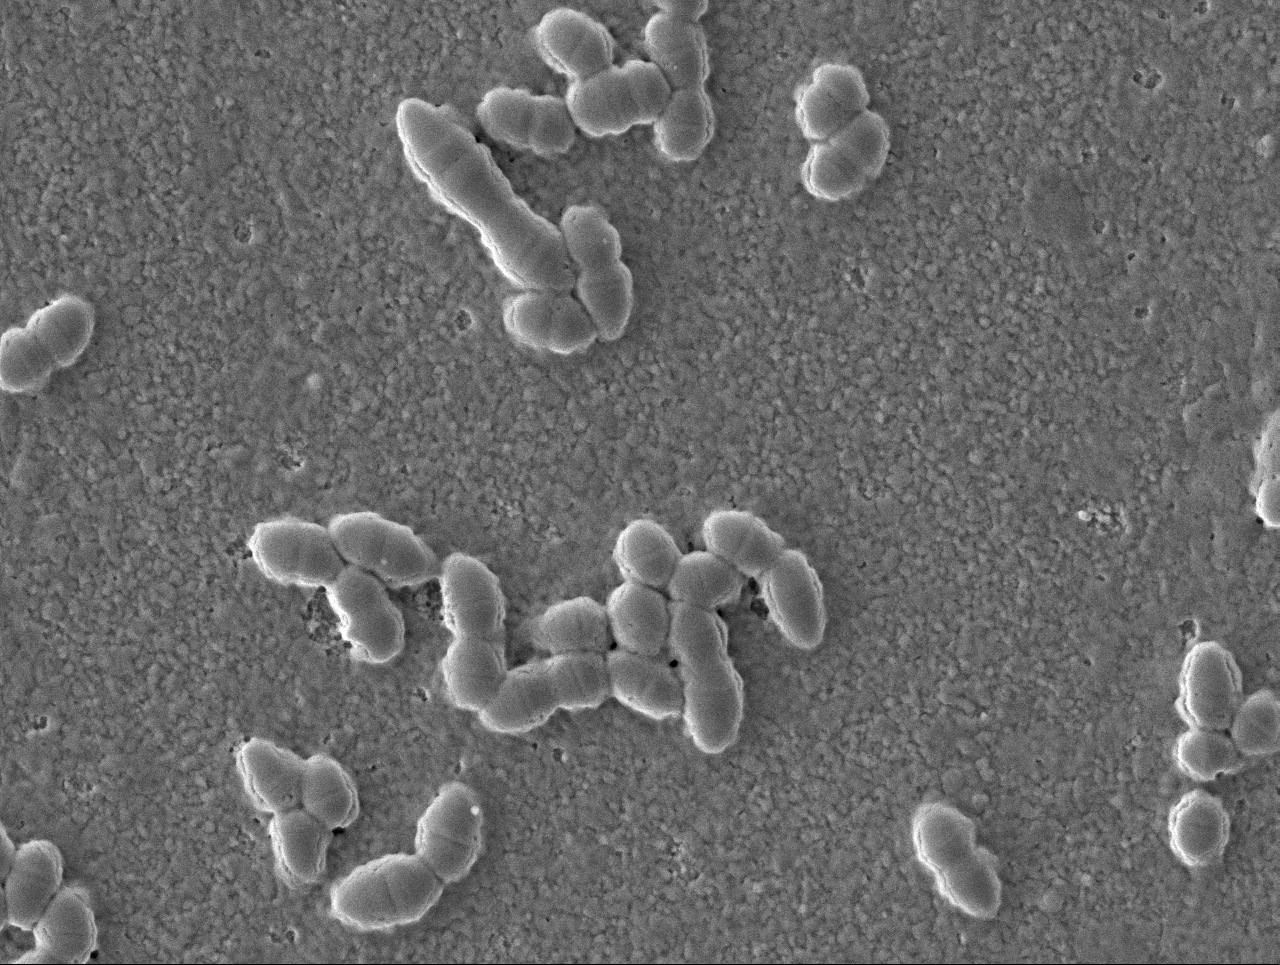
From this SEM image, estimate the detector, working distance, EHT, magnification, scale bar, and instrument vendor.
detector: LEI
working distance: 15.1 mm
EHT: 3 kV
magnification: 6 K X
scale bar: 1000 nm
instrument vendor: JEOL